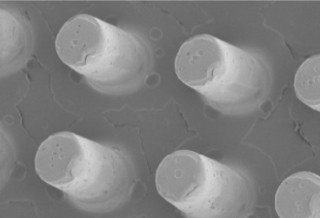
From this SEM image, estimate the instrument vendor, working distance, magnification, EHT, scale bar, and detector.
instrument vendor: Hitachi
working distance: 9.2 mm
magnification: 5 K X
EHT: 1 kV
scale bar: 10000 nm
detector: SE(M)